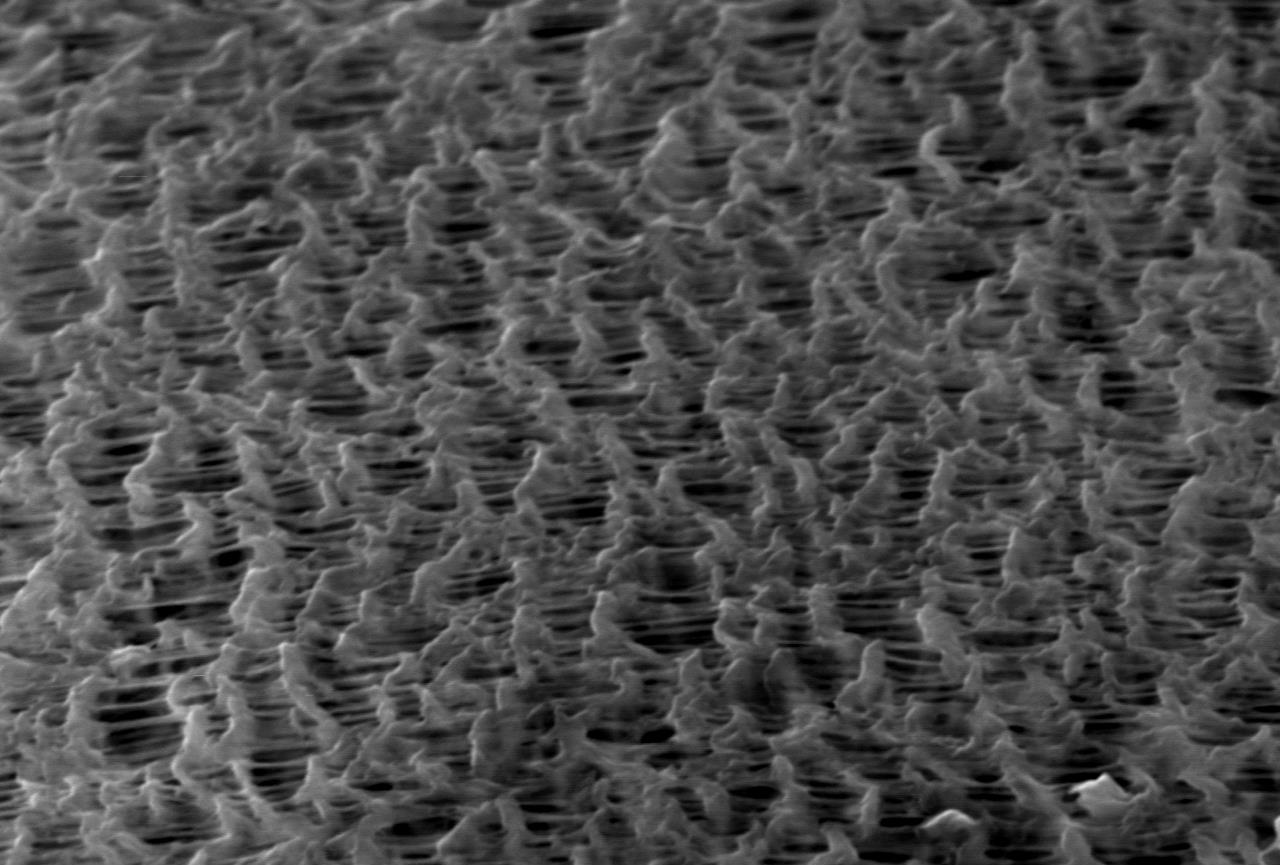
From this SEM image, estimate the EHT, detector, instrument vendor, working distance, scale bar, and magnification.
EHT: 20 kV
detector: SEI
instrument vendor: JEOL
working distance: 11 mm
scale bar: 5000 nm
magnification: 3 K X